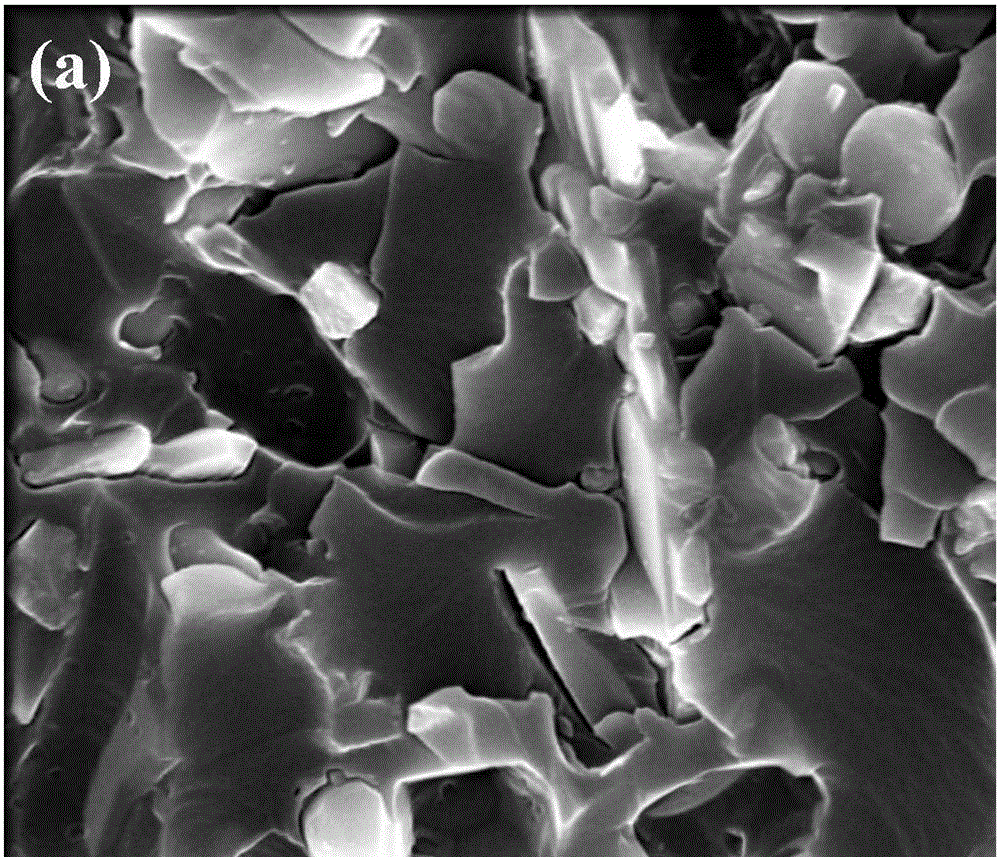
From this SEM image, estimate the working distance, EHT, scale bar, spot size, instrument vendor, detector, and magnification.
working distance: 5.4 mm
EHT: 10 kV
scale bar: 2000 nm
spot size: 3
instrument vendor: FEI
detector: TLD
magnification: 50 K X